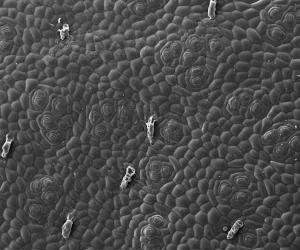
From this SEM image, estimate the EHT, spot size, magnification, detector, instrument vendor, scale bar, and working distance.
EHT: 20 kV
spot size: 3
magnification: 0.25 K X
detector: ETD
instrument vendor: FEI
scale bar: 500000 nm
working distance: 10.4 mm